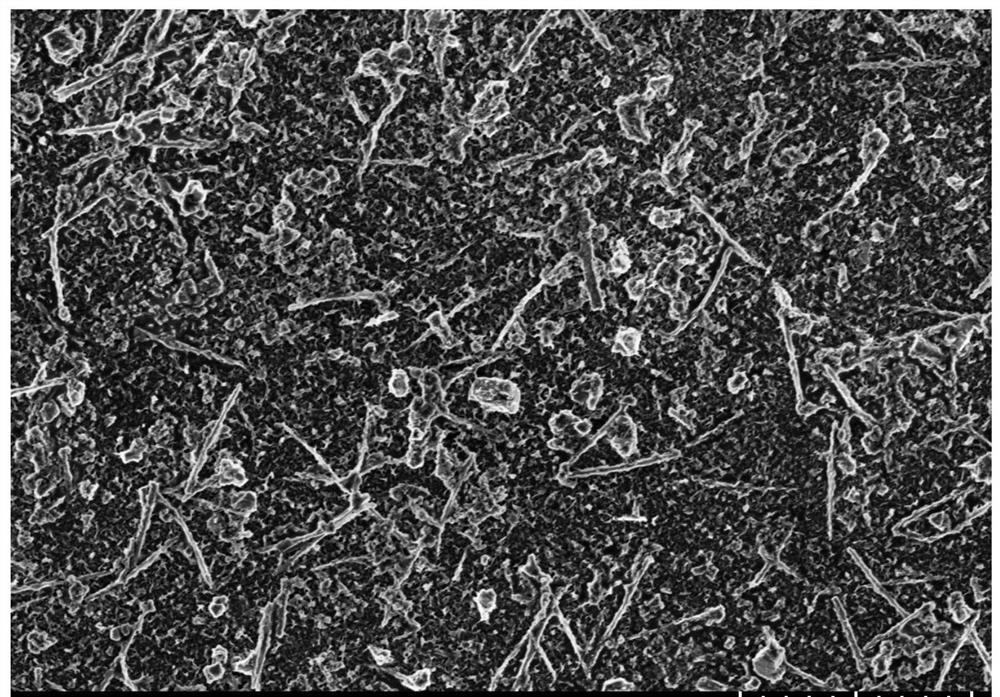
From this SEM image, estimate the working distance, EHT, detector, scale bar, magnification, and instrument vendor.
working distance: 10.6 mm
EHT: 3 kV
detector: SE(UL)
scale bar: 10000 nm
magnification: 3 K X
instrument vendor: Hitachi